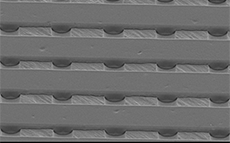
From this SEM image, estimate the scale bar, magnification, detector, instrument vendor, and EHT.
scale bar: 50000 nm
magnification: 0.5 K X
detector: SEI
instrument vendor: JEOL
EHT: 10 kV